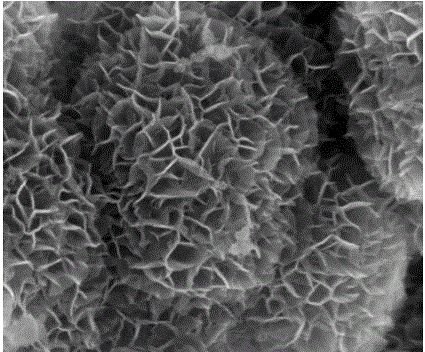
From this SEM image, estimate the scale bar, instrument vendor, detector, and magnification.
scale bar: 500 nm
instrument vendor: FEI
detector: TLD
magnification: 200 K X